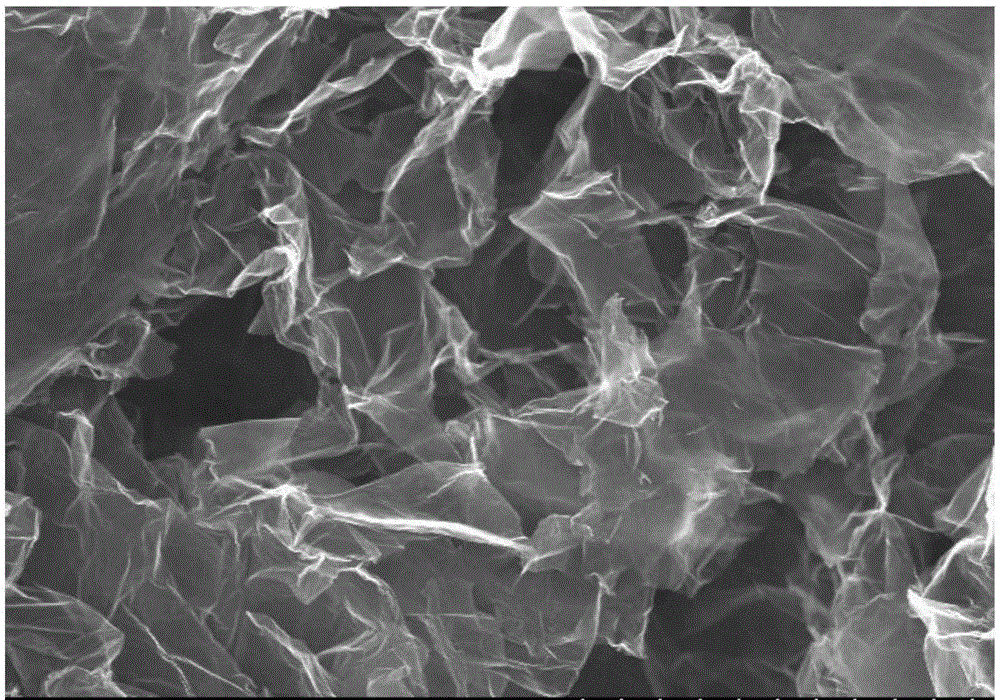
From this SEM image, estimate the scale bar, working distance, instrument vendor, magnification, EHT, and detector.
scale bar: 5000 nm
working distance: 10.5 mm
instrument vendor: Hitachi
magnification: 10 K X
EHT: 15 kV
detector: SE(U)